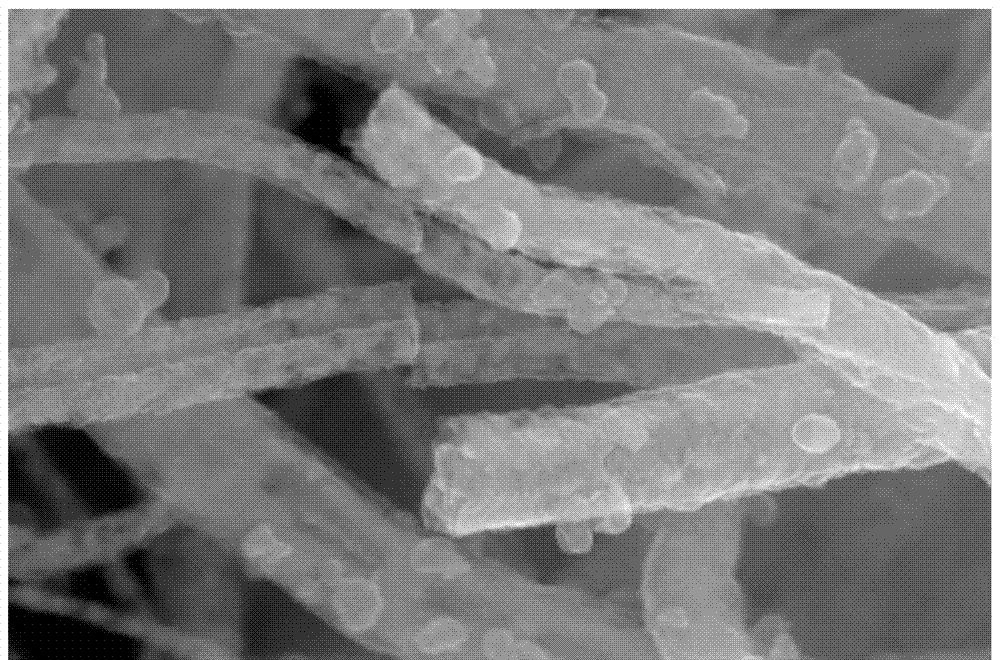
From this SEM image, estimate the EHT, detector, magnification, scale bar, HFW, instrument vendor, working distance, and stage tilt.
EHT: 15 kV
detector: TLD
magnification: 100 K X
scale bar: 300 nm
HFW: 1.27 µm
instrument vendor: FEI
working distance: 4 mm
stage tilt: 0°